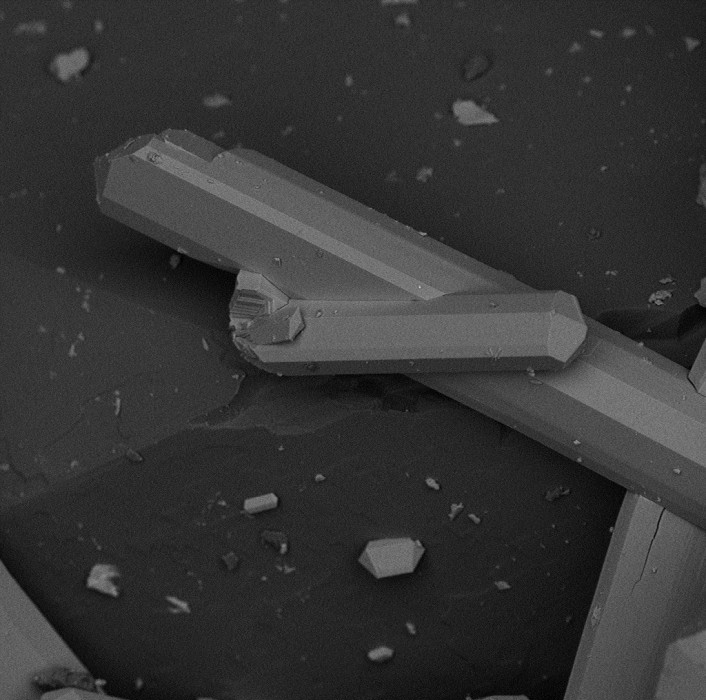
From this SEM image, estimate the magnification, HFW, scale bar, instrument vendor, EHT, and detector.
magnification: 1 K X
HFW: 271 µm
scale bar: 80000 nm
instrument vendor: other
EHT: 10 kV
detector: BSD Full